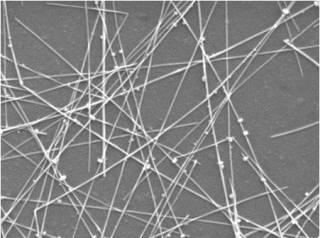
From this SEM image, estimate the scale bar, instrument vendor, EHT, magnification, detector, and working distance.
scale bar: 1000 nm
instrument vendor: JEOL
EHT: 5 kV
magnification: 20 K X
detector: SEI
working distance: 10.3 mm